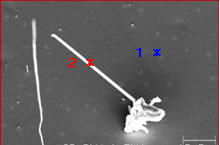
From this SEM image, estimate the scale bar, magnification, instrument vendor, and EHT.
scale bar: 10000 nm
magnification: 1.5 K X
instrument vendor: JEOL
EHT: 20 kV